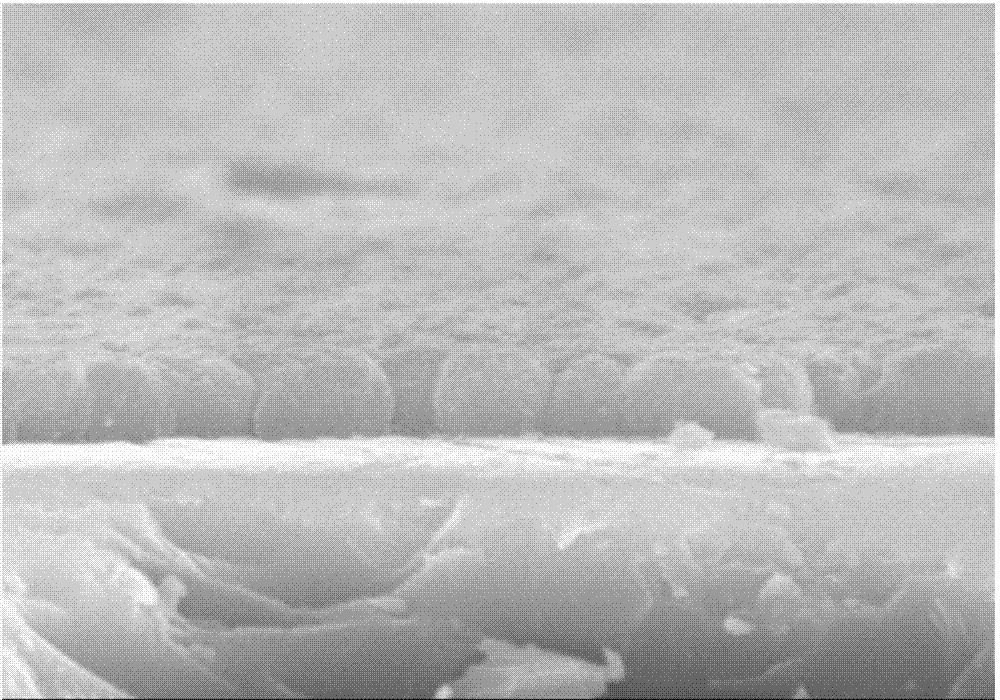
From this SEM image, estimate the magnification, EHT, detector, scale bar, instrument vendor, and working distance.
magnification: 20 K X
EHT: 15 kV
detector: SE(M)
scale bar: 2000 nm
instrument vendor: Hitachi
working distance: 13.8 mm